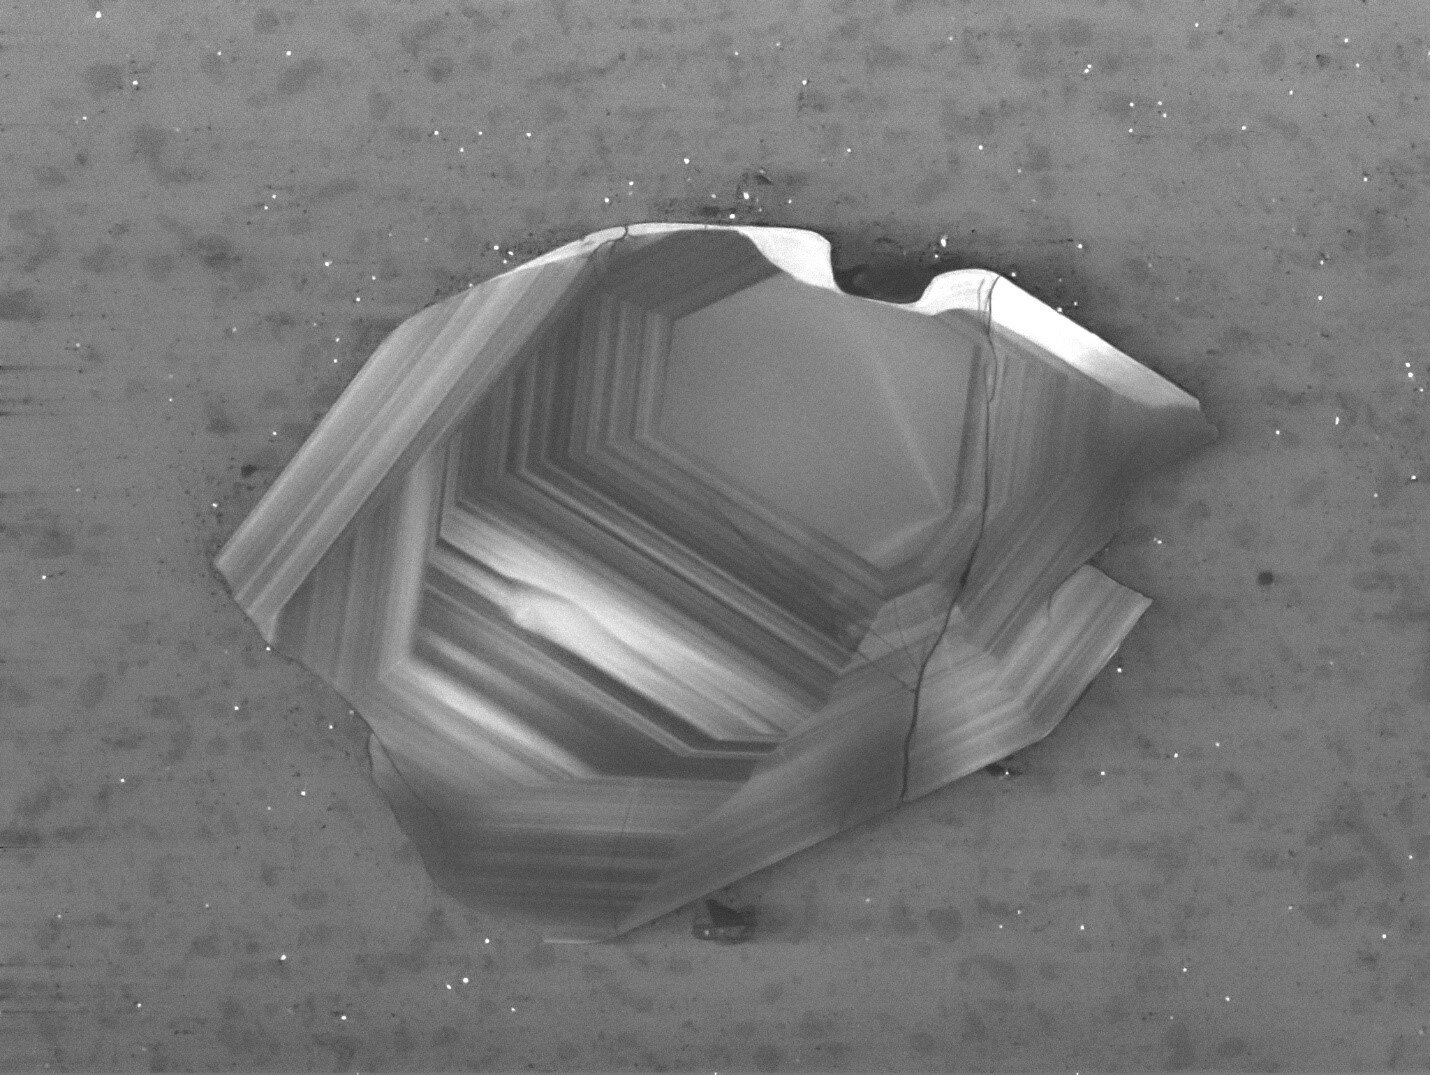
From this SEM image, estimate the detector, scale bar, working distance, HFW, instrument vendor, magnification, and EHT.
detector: CL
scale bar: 100000 nm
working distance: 17.38 mm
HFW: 395 µm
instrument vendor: Tescan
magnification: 1.4 K X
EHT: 10 kV